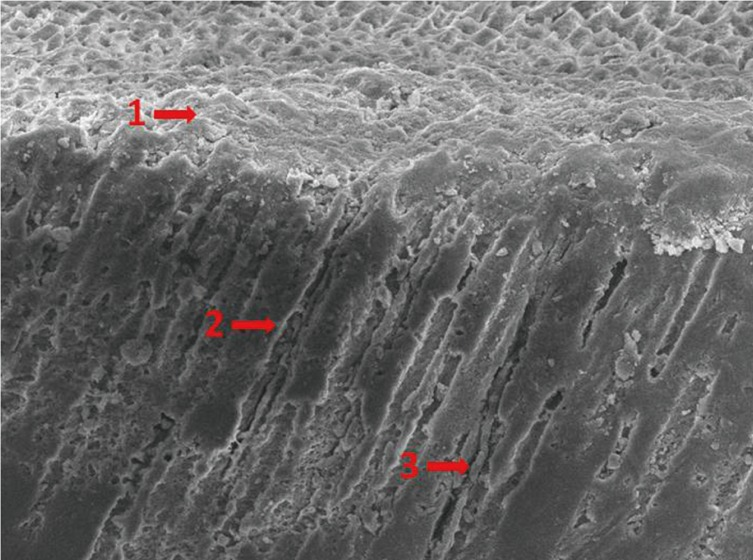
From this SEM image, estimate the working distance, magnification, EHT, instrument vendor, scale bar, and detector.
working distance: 10 mm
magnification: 1 K X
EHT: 10 kV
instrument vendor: JEOL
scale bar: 10000 nm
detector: SEI